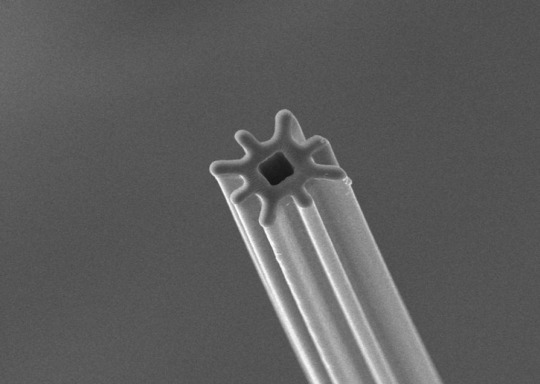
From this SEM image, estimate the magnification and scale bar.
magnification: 1 K X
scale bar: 10000 nm